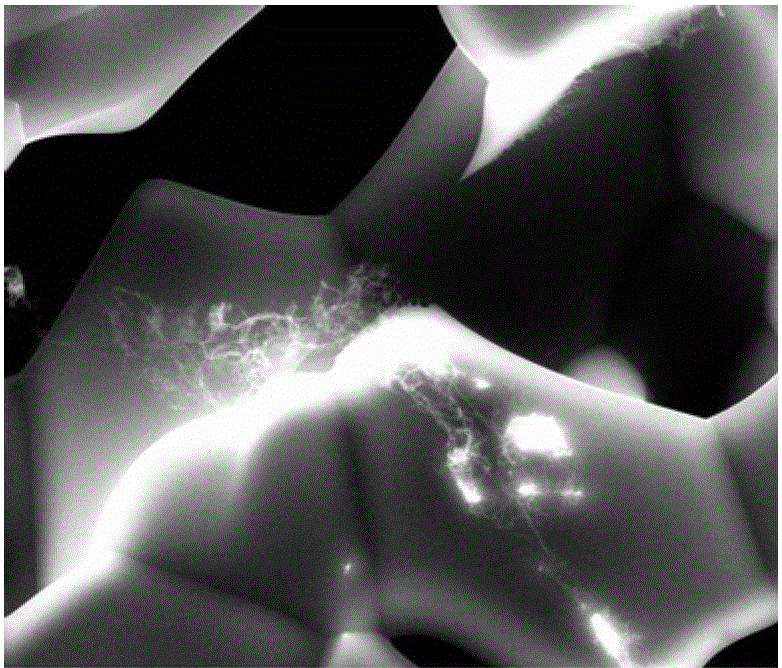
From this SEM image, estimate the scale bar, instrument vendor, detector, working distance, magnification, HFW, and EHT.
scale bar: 50000 nm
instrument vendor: FEI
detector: GSED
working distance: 12.1 mm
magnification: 2 K X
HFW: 128 µm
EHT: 20 kV